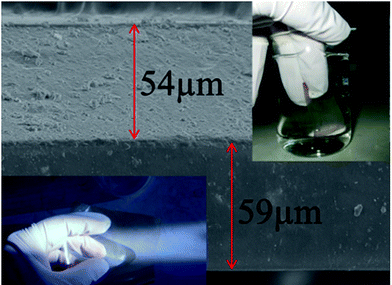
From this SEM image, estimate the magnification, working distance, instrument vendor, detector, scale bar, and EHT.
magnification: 0.6 K X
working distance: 9.9 mm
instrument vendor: JEOL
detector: SEI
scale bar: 10000 nm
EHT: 5 kV